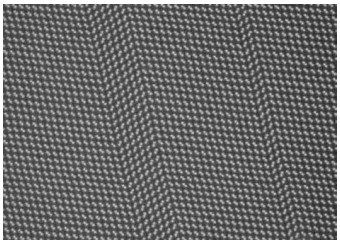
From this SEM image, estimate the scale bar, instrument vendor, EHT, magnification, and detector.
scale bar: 3 nm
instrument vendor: Hitachi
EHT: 200 kV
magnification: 8000 K X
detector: ZC